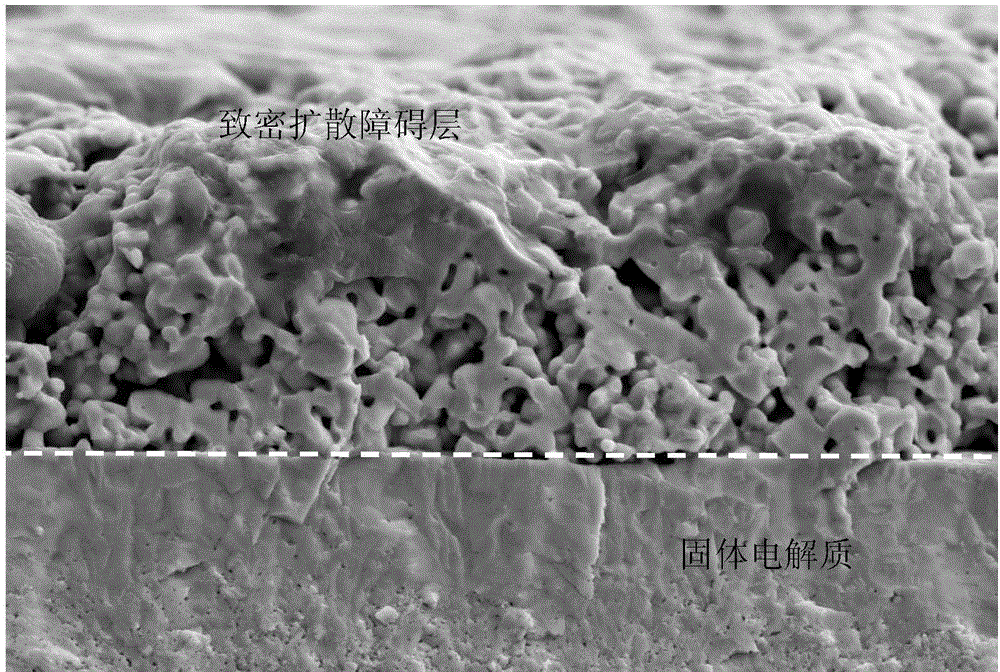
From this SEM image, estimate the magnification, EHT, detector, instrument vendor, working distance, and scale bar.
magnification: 5 K X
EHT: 15 kV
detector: SE2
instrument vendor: Zeiss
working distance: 9 mm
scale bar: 2000 nm